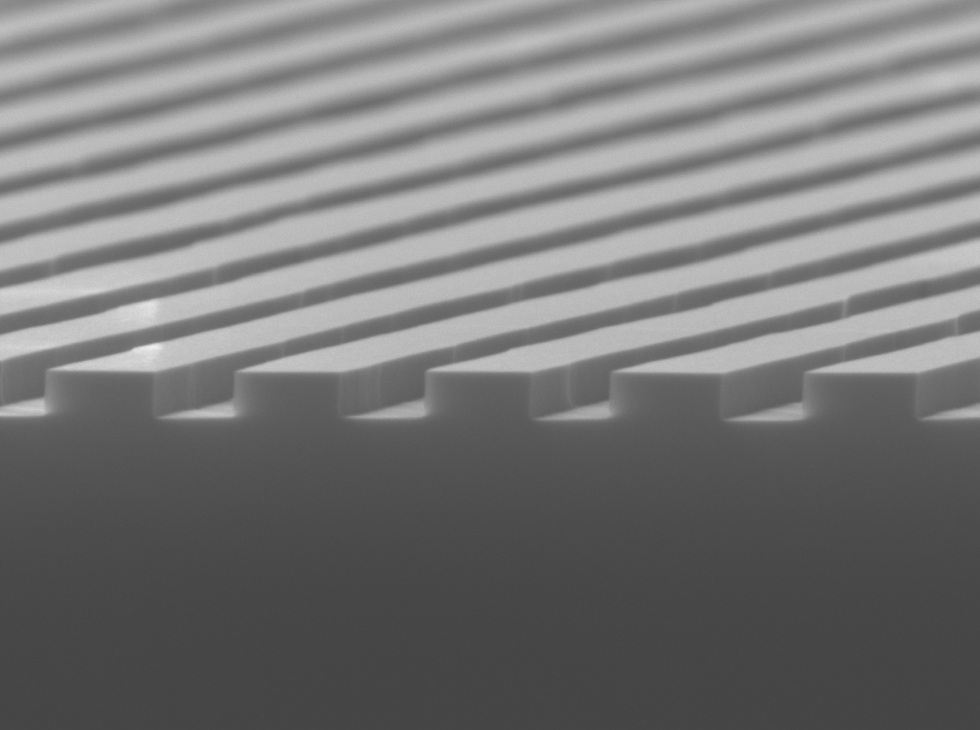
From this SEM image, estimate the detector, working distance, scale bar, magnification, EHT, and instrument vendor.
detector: SEI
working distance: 7.5 mm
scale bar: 100 nm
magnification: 43 K X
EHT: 8 kV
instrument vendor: JEOL